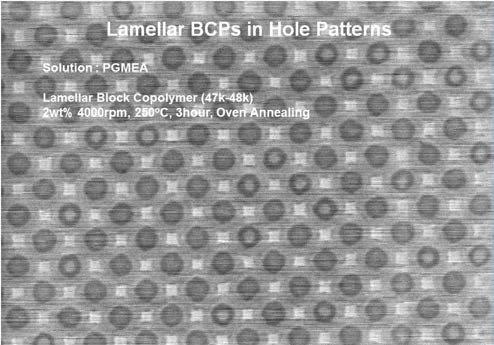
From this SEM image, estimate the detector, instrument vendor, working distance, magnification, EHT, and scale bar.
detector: SE(U)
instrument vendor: Hitachi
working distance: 3 mm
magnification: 50 K X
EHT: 1 kV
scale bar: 1000 nm